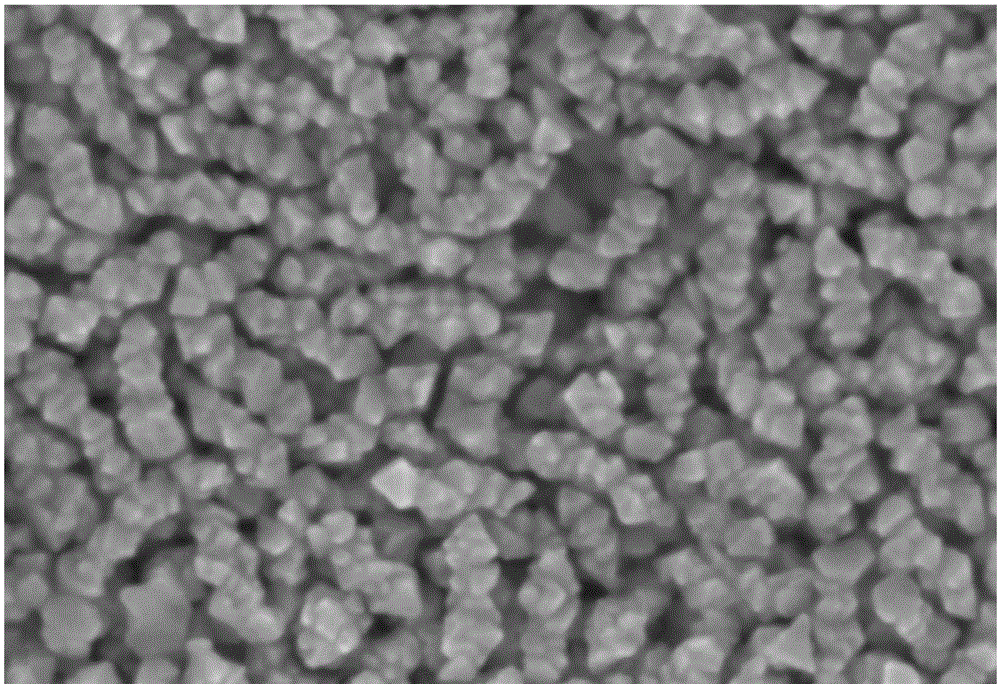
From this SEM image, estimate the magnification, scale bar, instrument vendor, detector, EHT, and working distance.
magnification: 150 K X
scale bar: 20 nm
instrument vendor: Zeiss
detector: InLens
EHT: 5 kV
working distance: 3 mm